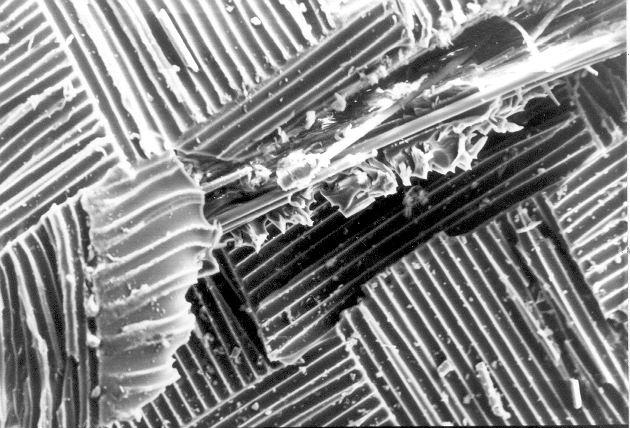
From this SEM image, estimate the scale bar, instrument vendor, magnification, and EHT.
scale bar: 100000 nm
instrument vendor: JEOL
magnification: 0.1 K X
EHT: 25 kV